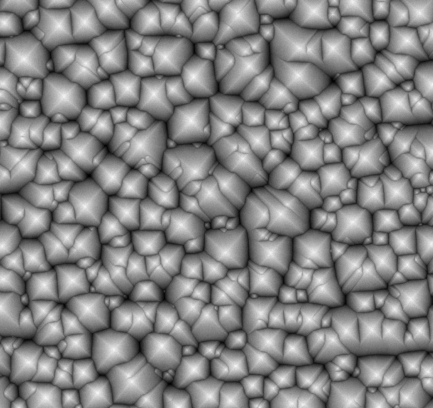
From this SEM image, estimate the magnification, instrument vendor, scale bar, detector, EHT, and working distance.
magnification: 10 K X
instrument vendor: other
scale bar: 5000 nm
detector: BSE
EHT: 15 kV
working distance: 5.5 mm